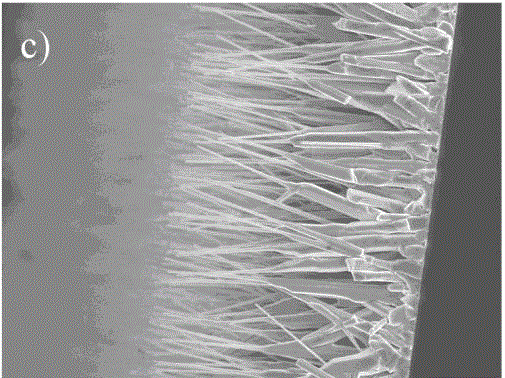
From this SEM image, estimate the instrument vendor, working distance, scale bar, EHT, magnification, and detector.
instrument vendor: JEOL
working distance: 11.7 mm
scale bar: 10000 nm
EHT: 10 kV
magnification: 2 K X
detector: SEI